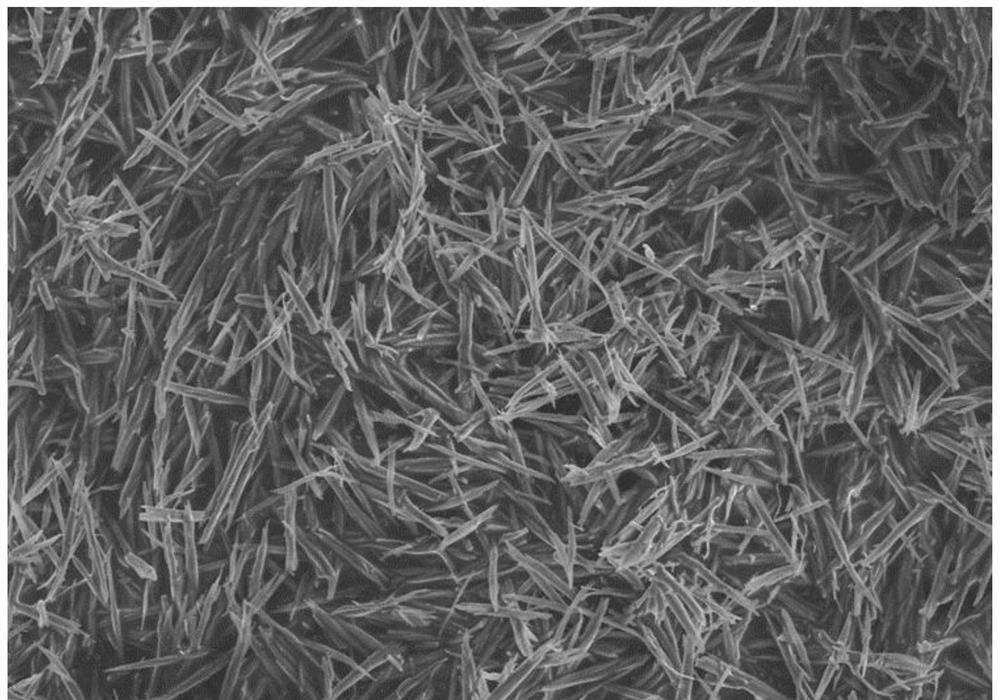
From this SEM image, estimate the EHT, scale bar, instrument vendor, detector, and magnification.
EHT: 5 kV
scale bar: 20000 nm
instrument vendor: Hitachi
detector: SE(UL)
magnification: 4.5 K X